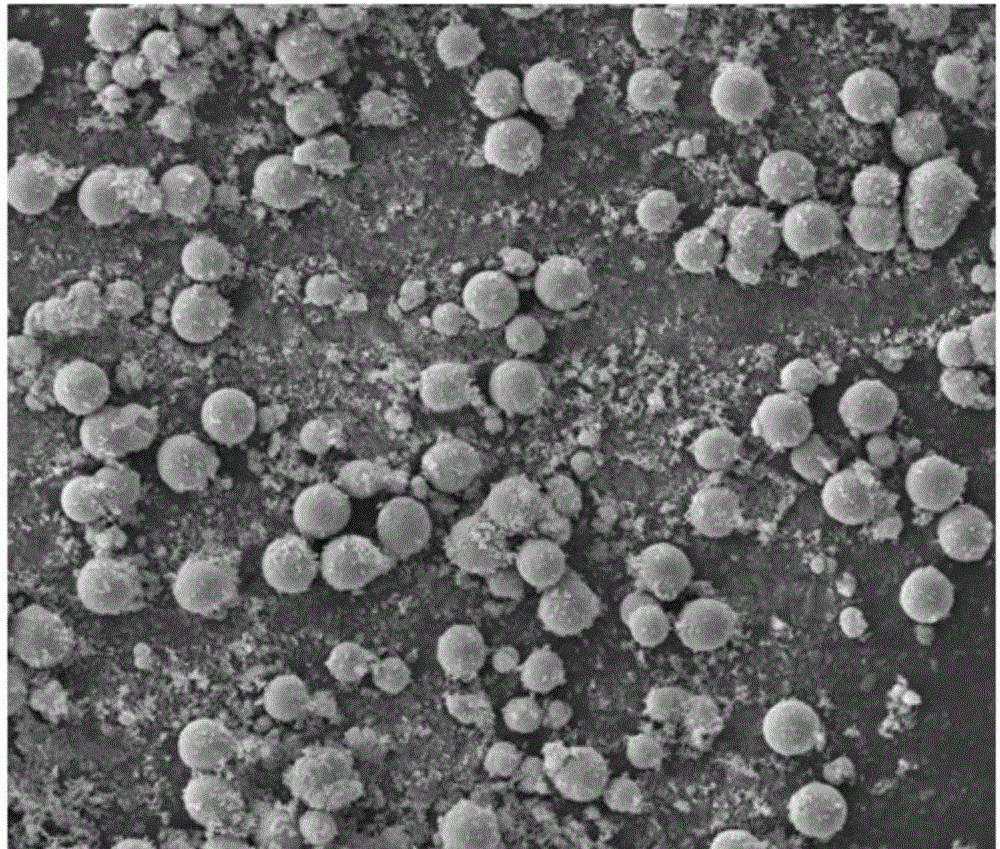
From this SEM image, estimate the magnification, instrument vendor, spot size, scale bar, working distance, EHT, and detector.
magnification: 1 K X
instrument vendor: FEI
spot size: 5.5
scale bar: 20000 nm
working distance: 11.4 mm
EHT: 15 kV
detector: ETD